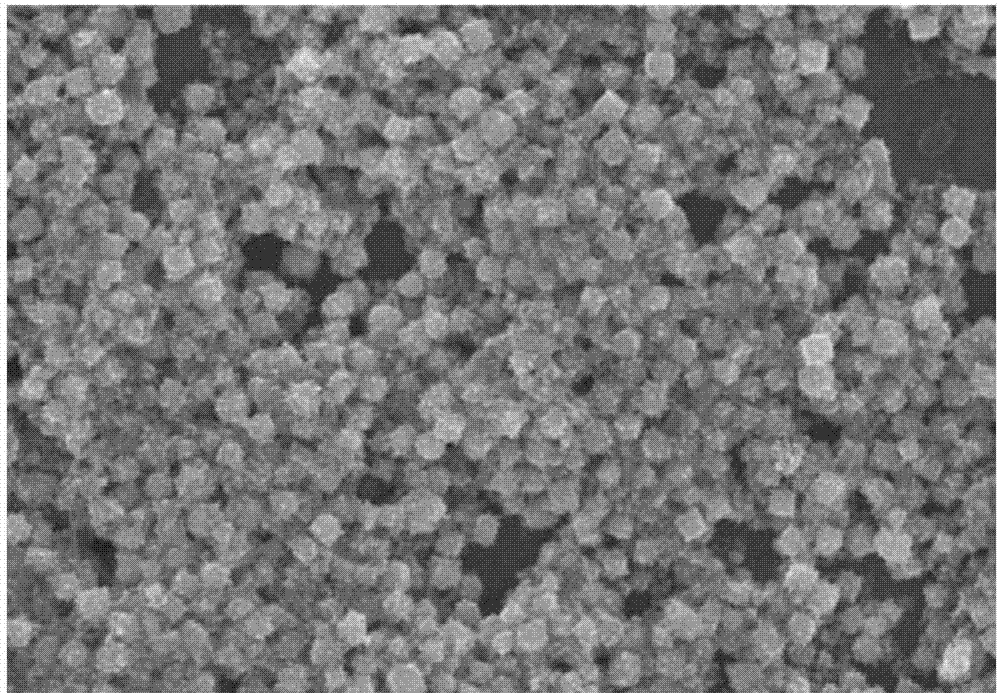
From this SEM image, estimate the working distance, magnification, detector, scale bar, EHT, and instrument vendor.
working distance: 6.6 mm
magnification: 22 K X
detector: SE(U)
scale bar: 2000 nm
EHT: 10 kV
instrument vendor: Hitachi